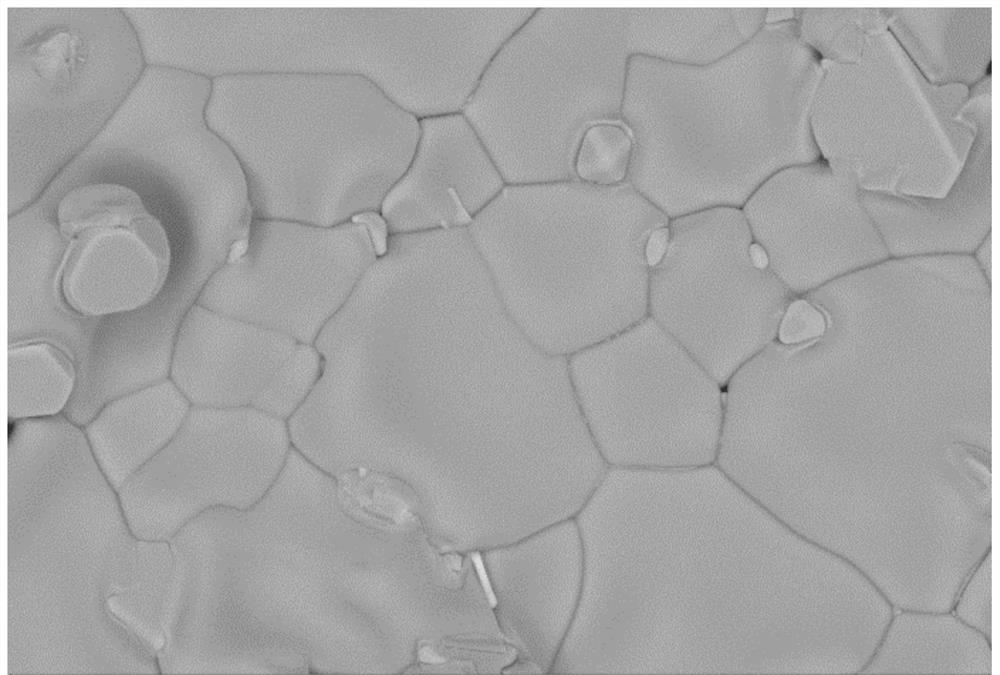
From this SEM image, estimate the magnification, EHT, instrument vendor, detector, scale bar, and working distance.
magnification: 5 K X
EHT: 15 kV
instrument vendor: Zeiss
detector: AsB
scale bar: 2000 nm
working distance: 8.4 mm